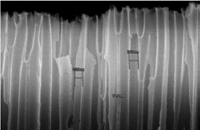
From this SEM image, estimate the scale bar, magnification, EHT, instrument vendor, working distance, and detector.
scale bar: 2000 nm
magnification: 22 K X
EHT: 15 kV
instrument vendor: Hitachi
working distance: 9.6 mm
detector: SE(U)